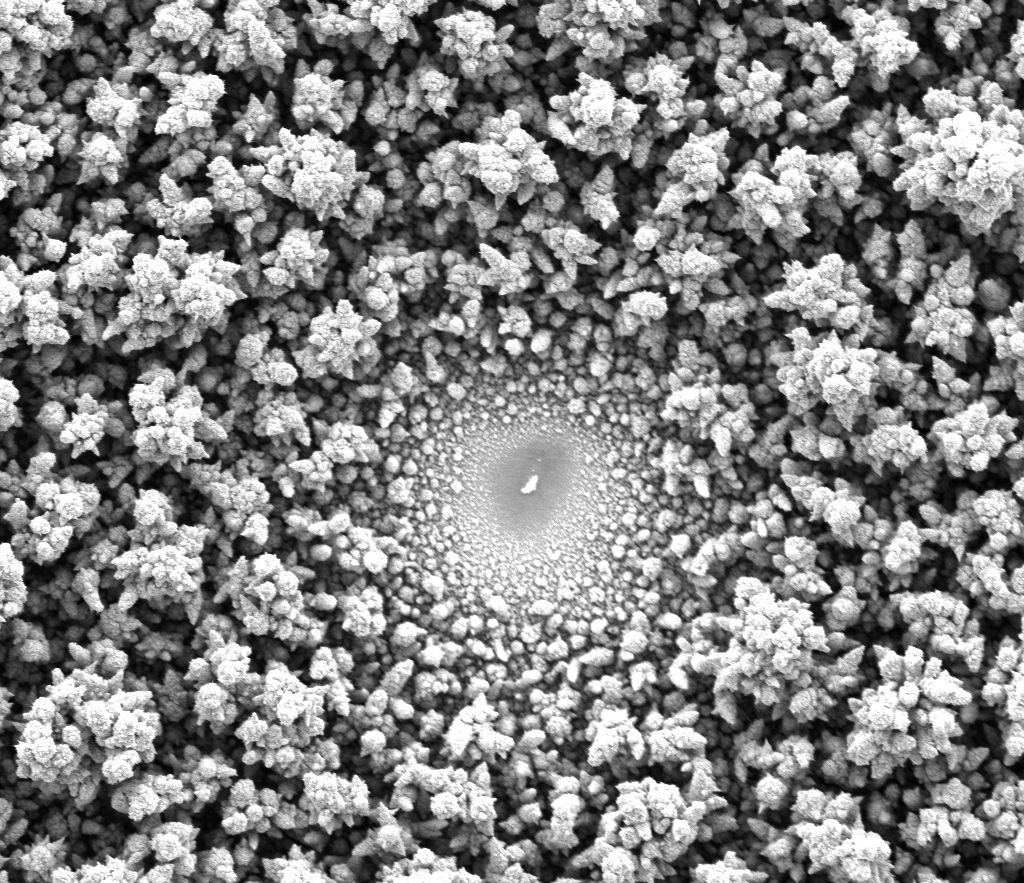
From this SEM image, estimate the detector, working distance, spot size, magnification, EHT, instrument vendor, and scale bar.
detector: SE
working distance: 10 mm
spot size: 3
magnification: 2.861 K X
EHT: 10 kV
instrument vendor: FEI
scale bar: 40000 nm